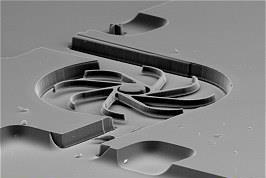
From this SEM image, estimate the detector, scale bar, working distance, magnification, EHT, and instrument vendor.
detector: SEI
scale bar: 200000 nm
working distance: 48 mm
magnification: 0.1 K X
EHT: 25 kV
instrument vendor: Tescan